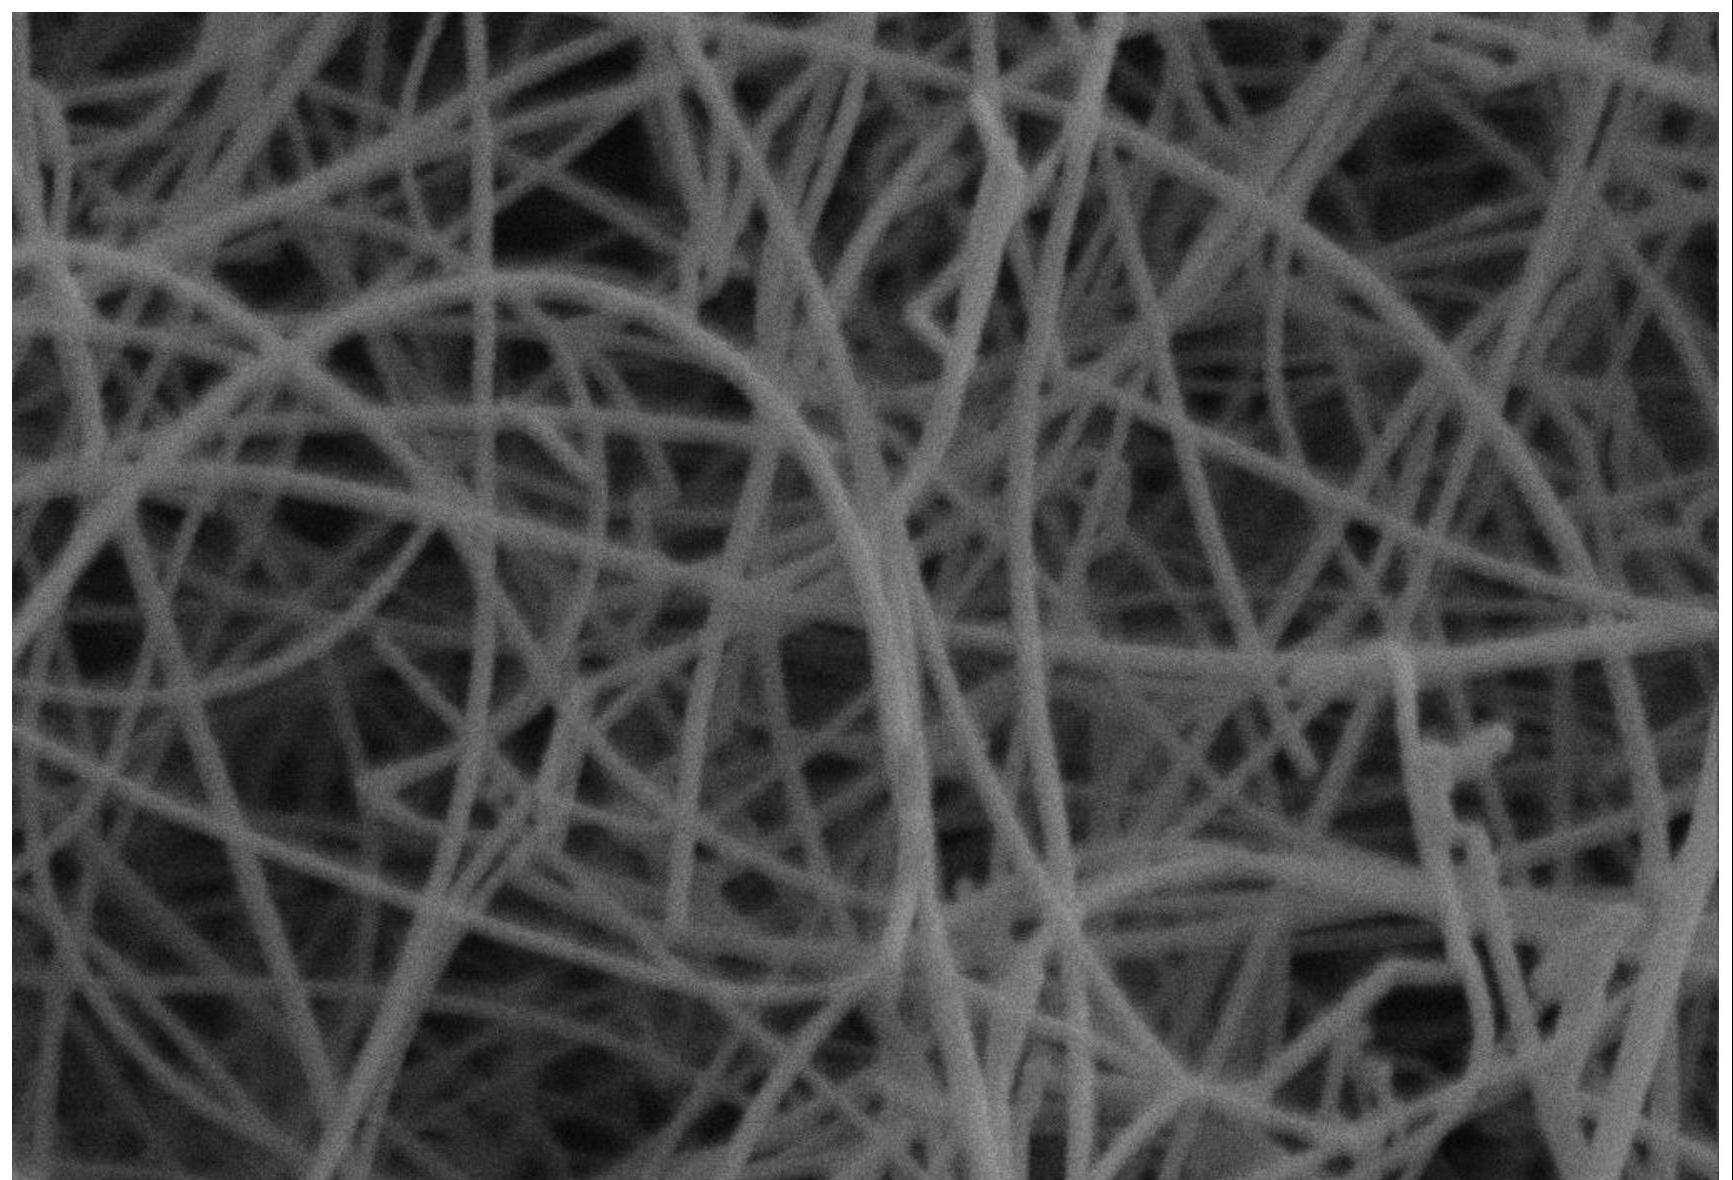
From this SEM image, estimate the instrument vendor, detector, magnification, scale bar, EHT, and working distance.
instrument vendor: other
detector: SE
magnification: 20 K X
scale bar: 1000 nm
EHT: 20 kV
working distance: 9.1 mm